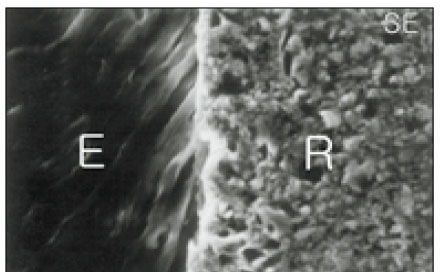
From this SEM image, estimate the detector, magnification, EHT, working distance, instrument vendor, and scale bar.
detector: SE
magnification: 1.5 K X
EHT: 20 kV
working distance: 39 mm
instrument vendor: JEOL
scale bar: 10000 nm